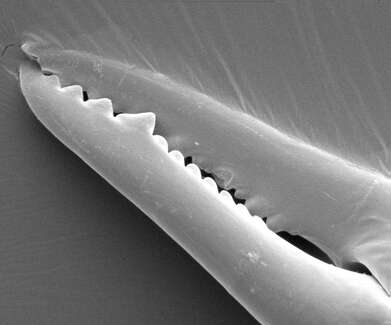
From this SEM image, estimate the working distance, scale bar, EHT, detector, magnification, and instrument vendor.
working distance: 11.1 mm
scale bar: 20000 nm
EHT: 5 kV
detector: ETD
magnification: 0.48 K X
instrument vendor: FEI